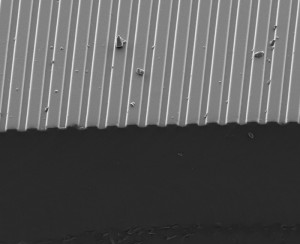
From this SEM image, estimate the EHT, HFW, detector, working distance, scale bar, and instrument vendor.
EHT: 5 kV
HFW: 150 µm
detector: ETD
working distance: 10 mm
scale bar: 50000 nm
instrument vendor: FEI